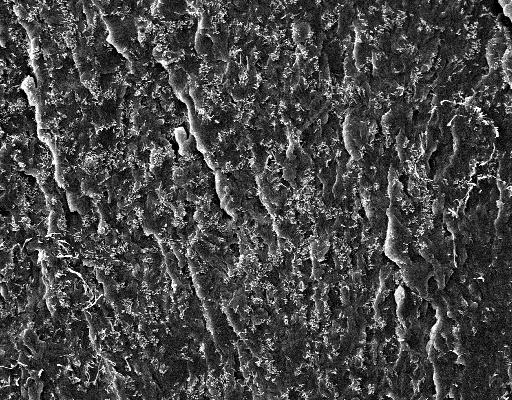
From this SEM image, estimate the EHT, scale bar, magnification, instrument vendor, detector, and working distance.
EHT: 10 kV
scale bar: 30000 nm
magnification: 1.095 K X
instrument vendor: Zeiss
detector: SE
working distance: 5.2 mm